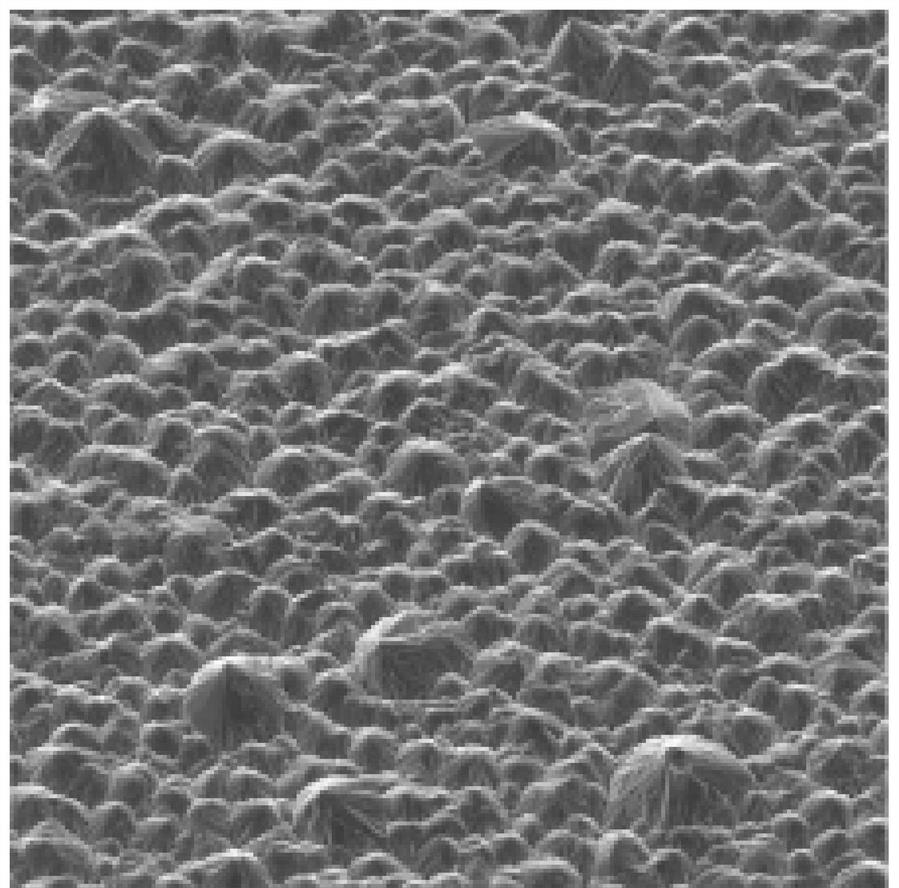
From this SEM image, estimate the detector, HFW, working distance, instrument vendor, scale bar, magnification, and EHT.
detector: SE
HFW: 208 µm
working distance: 22.01 mm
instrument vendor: Tescan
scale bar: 50000 nm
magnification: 1 K X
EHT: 20 kV